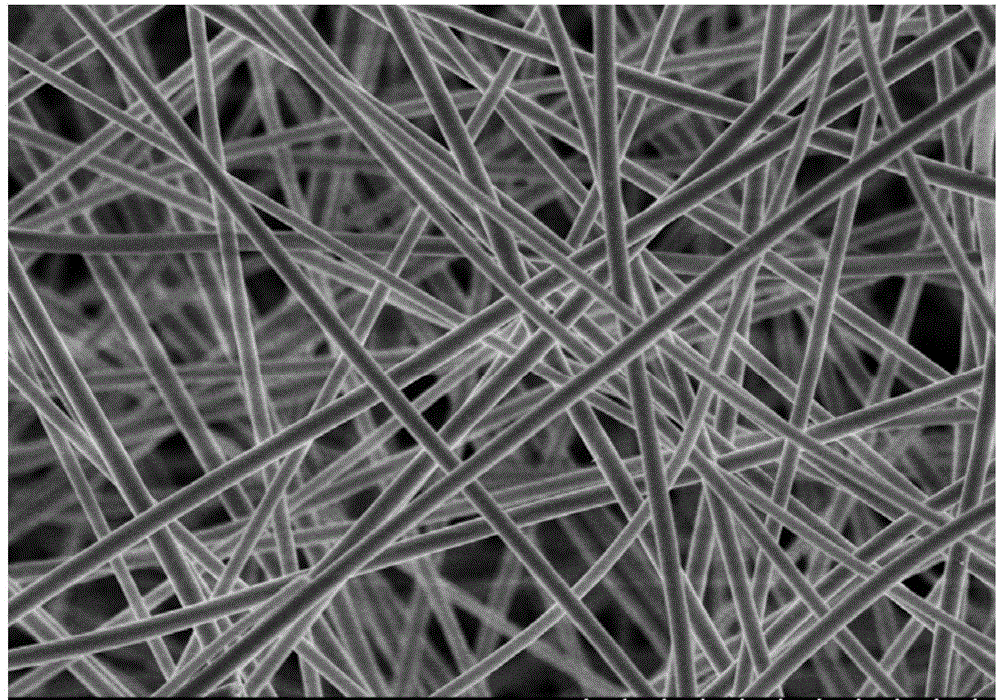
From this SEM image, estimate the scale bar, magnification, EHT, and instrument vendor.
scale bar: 5000 nm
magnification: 10 K X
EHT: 5 kV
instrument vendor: Hitachi